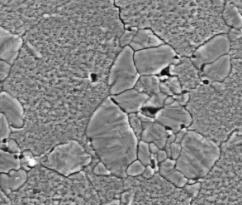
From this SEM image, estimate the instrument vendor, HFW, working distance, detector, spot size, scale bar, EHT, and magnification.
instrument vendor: FEI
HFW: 29.8 µm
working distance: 6.5 mm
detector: LVD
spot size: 4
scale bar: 10000 nm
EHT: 10 kV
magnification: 10 K X